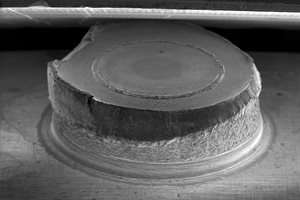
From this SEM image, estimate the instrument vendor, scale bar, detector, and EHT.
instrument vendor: Zeiss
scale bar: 1000000 nm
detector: SE1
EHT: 20 kV